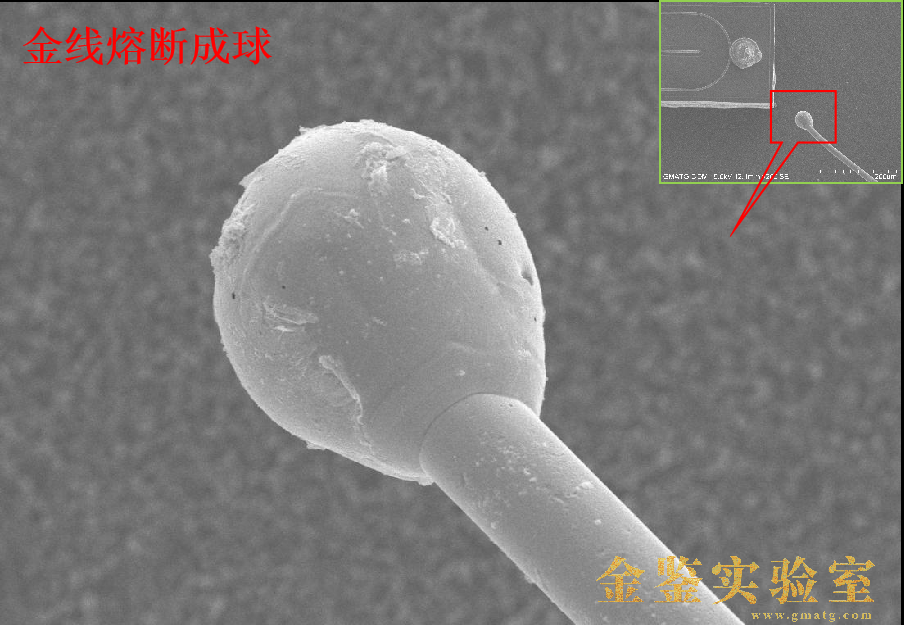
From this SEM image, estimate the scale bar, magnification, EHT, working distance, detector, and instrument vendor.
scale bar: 50000 nm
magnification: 1 K X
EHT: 15 kV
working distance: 12.1 mm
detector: SE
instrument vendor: Hitachi